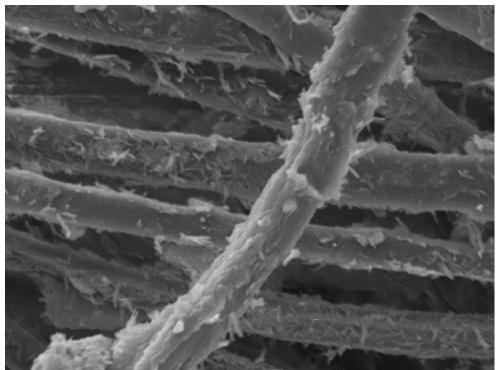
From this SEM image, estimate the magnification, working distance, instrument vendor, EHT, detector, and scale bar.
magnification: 5 K X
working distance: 11.88 mm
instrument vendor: Tescan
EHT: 10 kV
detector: SE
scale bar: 10000 nm